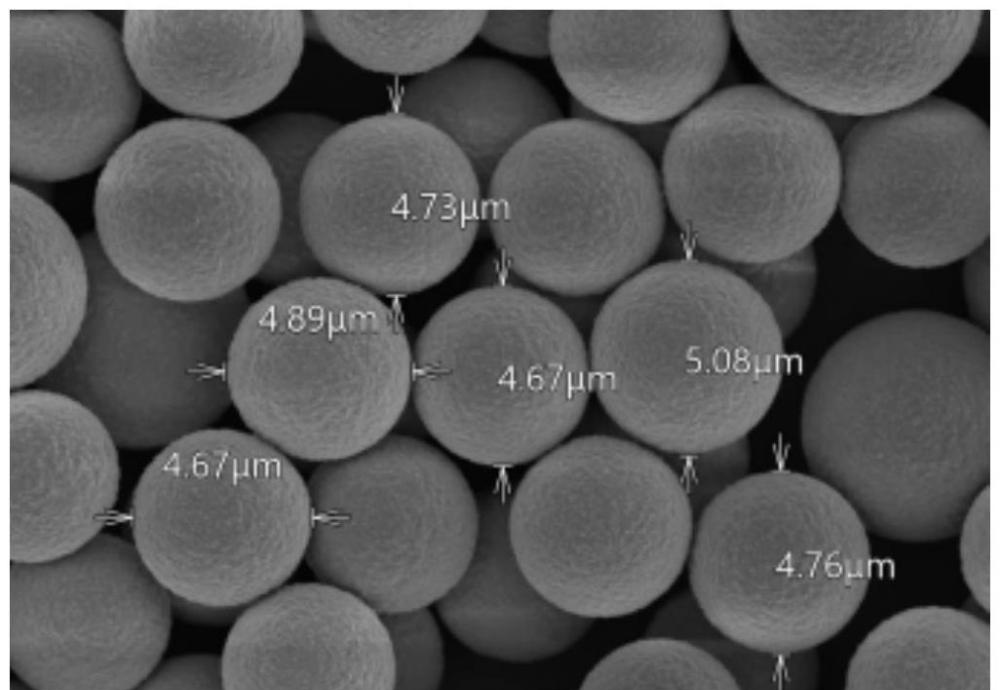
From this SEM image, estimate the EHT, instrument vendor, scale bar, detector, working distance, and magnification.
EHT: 5 kV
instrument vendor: Hitachi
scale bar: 10000 nm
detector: UD(+LD)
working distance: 8 mm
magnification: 5 K X